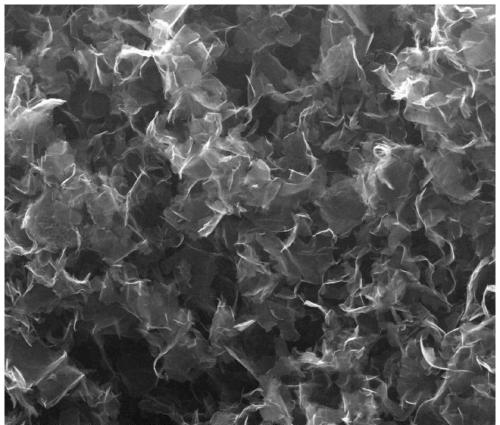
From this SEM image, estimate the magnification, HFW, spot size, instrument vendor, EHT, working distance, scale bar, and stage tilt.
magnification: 2.971 K X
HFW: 100 µm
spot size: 5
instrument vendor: FEI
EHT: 20 kV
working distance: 9.6 mm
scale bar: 30000 nm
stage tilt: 0°